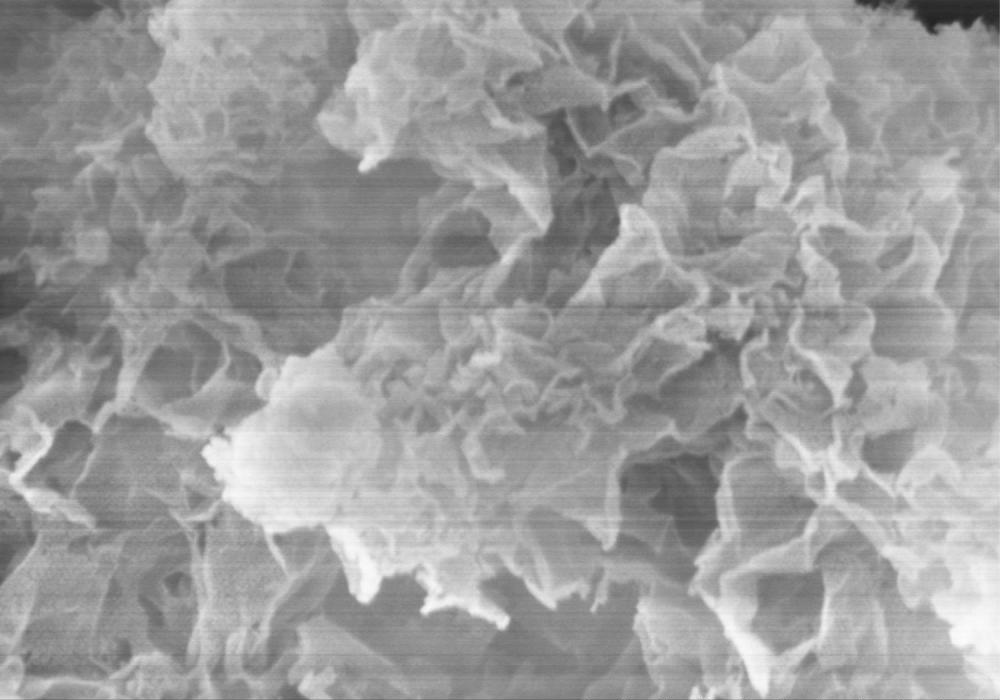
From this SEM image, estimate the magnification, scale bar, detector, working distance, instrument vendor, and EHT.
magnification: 50 K X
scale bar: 1000 nm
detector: SE(M)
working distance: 9 mm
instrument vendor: Hitachi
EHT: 5 kV